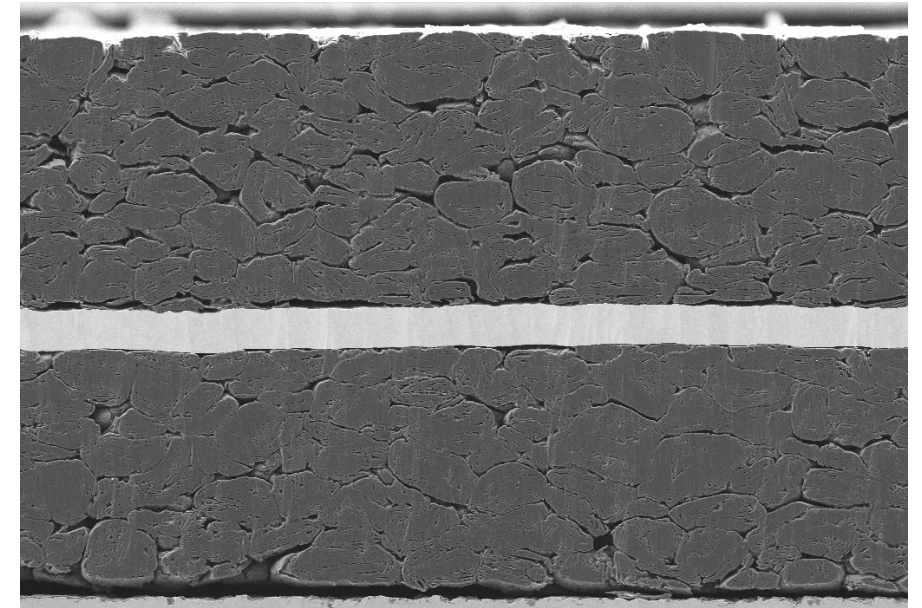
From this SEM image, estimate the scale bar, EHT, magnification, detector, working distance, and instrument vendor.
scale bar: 10000 nm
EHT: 5 kV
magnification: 0.55 K X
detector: SE2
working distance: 5.1 mm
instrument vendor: Zeiss